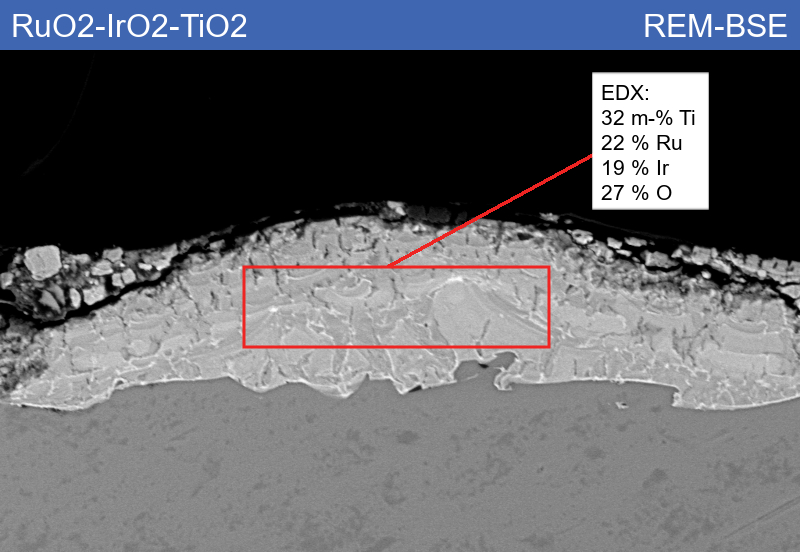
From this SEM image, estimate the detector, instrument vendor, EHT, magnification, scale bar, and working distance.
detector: HDBSD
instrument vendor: Zeiss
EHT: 15 kV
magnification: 1 K X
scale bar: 10000 nm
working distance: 8.5 mm